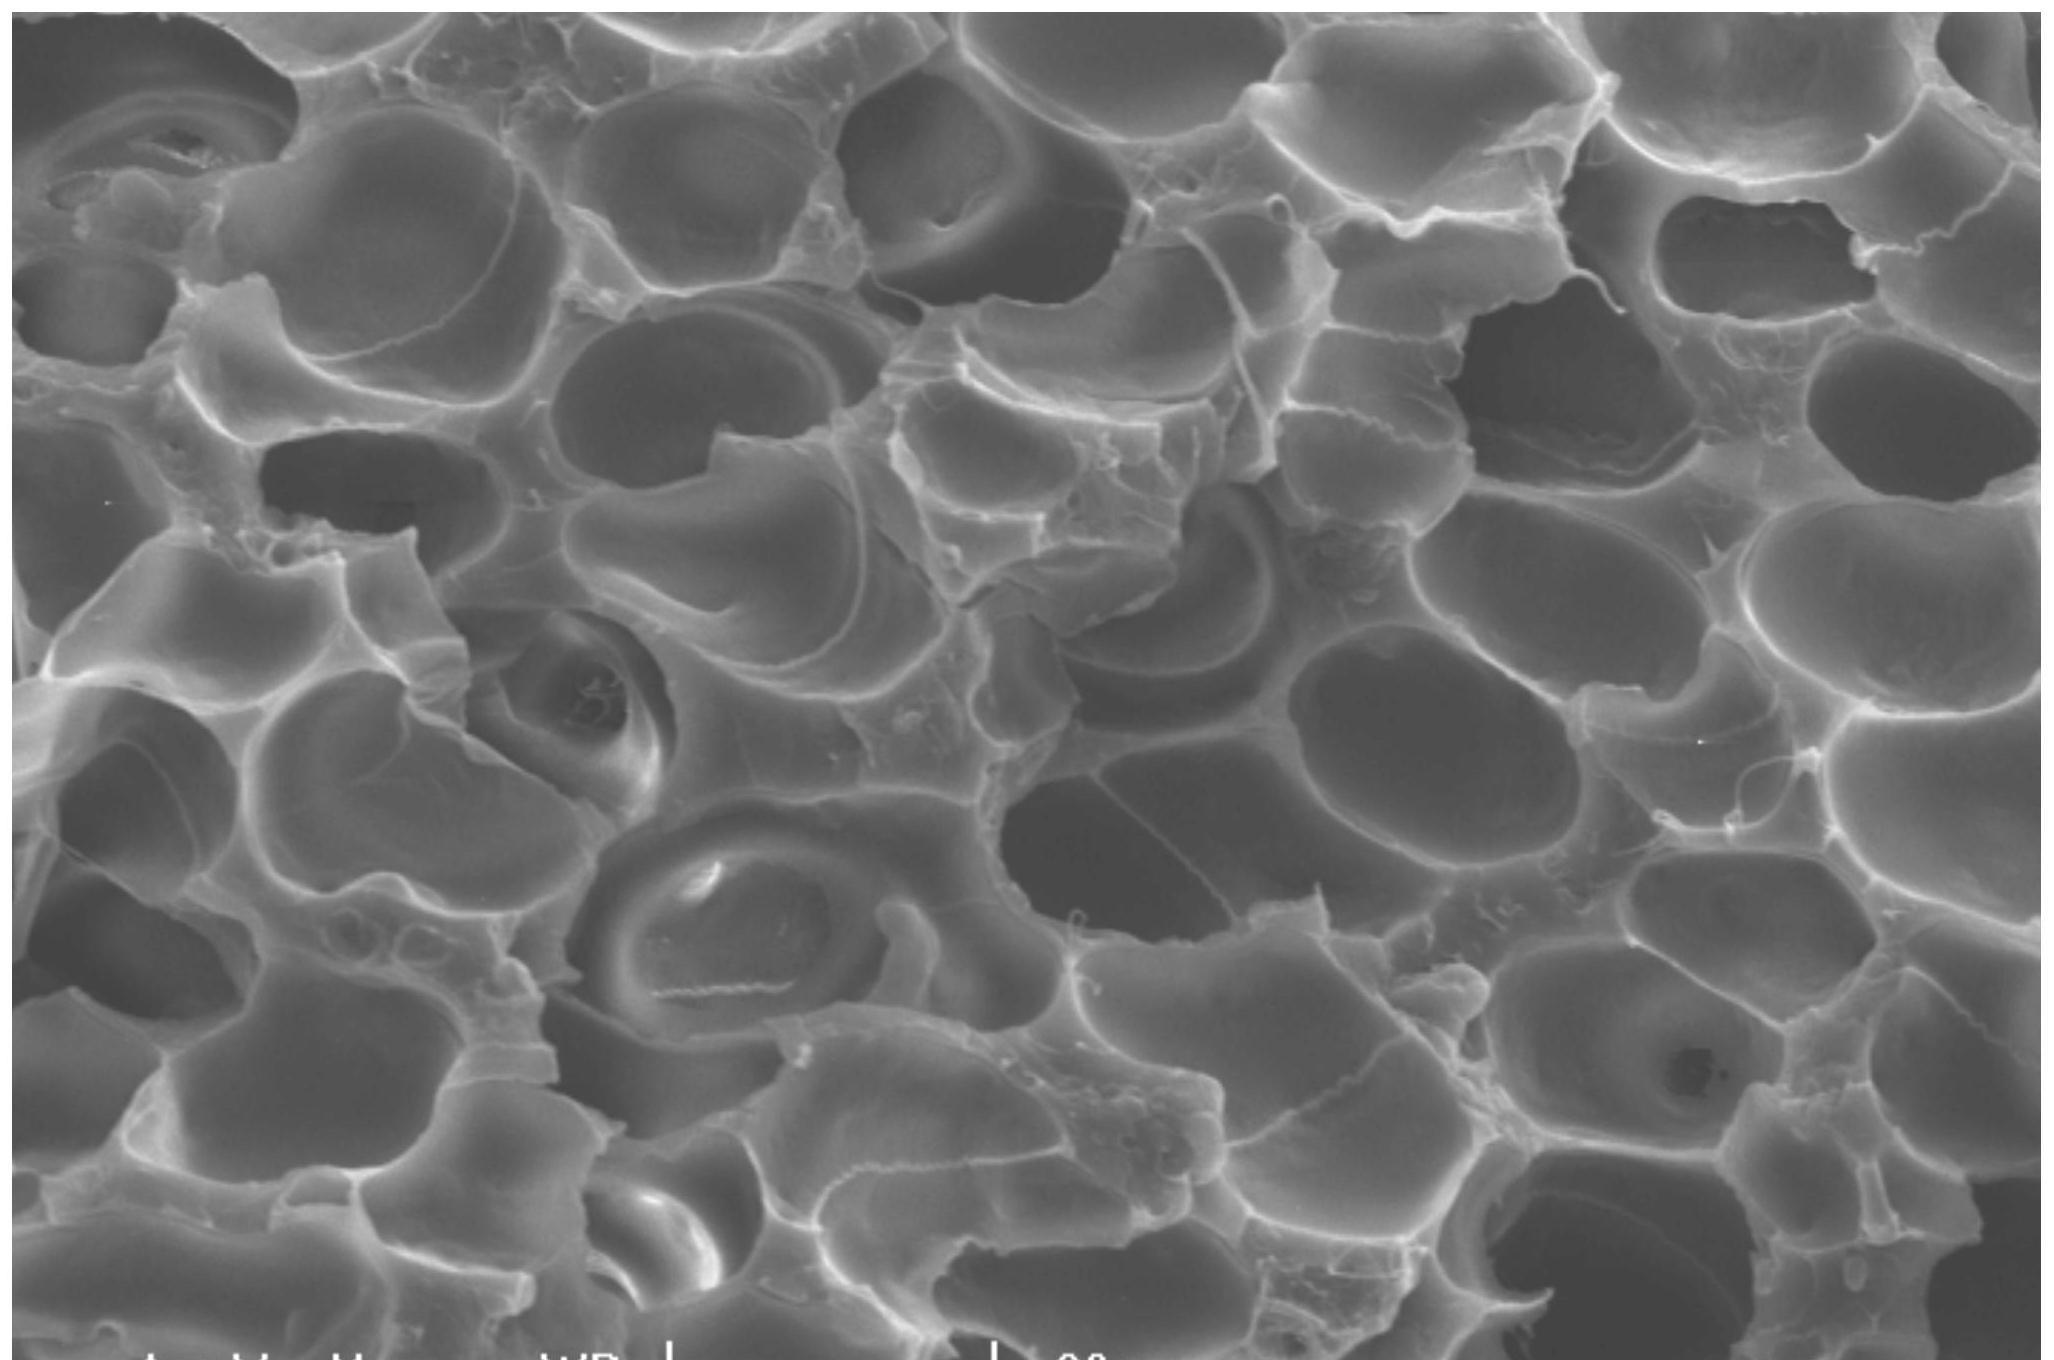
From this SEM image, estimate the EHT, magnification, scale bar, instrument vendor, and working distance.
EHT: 15 kV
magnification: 1 K X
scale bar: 20000 nm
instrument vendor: FEI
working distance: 20.8 mm